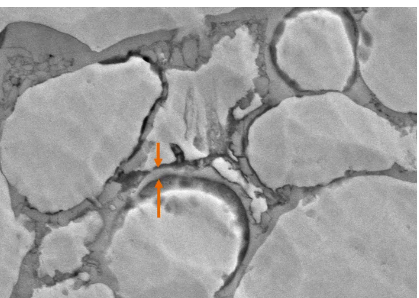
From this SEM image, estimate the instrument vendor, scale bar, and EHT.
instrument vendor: Zeiss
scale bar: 1000 nm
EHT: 10 kV